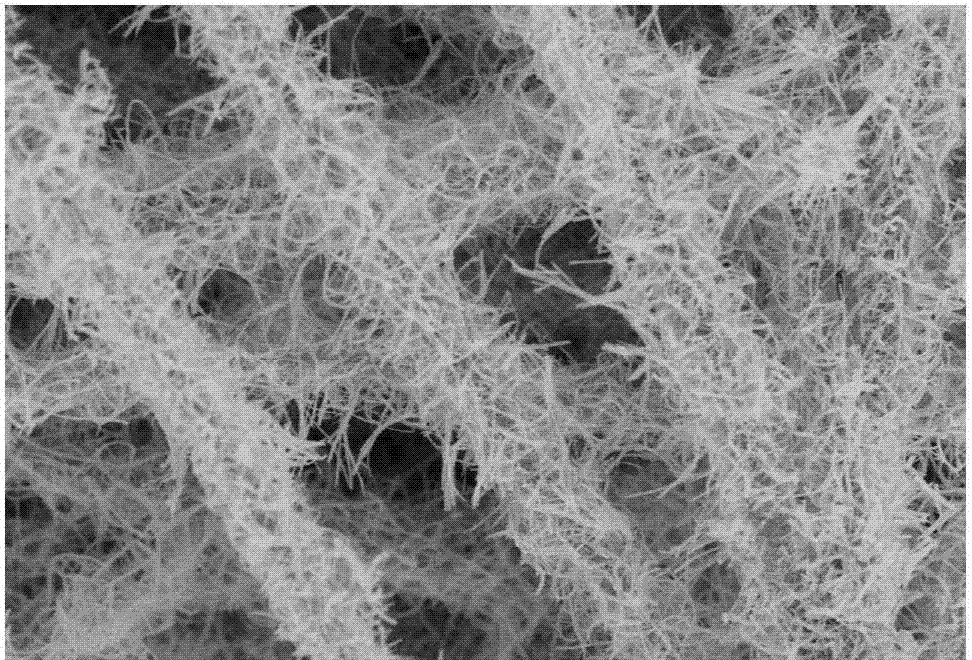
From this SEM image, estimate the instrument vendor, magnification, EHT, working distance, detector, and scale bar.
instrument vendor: Hitachi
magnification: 15 K X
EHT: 5 kV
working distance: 8.7 mm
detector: SE(U)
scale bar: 3000 nm